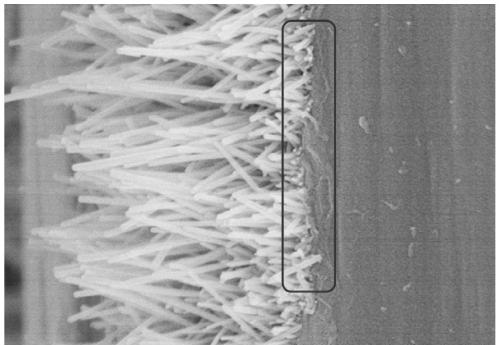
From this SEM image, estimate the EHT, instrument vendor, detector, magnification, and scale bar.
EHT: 5 kV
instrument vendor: Hitachi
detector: SE(M)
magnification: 50 K X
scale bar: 1000 nm